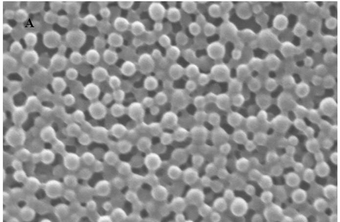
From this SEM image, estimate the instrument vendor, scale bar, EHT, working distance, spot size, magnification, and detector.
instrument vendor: Zeiss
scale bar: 300 nm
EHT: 20 kV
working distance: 9 mm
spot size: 224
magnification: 20.91 K X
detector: SE1